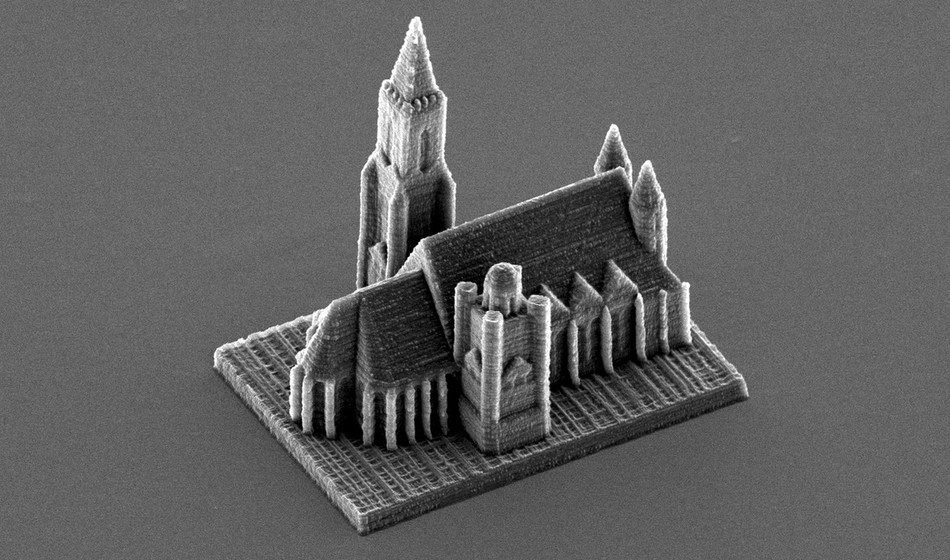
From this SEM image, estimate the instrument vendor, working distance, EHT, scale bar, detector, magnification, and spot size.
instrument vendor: FEI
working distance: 20.1 mm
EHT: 12 kV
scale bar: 50000 nm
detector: SE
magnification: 0.628 K X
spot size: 4